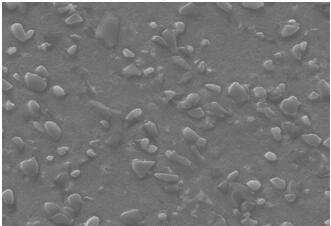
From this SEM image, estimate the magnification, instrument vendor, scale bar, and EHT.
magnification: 1 K X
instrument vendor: other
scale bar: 10000 nm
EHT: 25 kV